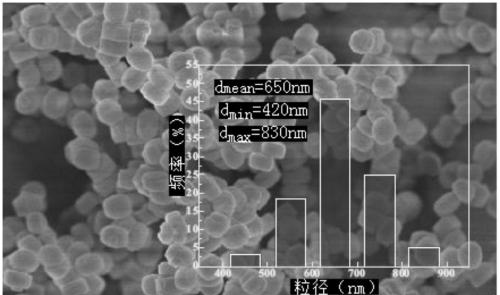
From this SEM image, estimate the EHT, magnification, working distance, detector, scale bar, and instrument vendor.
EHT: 5 kV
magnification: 9 K X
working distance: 4.5 mm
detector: SE(U)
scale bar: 5000 nm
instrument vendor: Hitachi